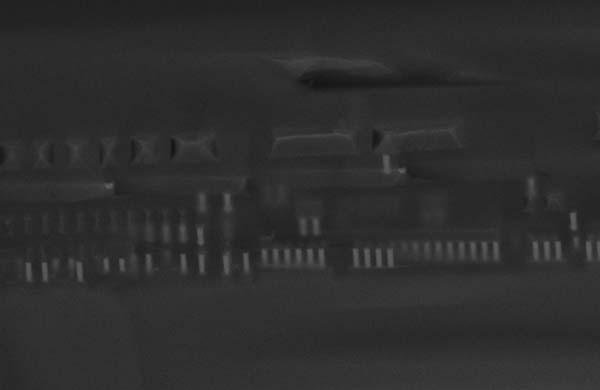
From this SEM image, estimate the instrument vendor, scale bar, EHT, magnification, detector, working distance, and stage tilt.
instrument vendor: Zeiss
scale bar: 2000 nm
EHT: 15 kV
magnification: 11.47 K X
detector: InLens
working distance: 4 mm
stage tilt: -15°